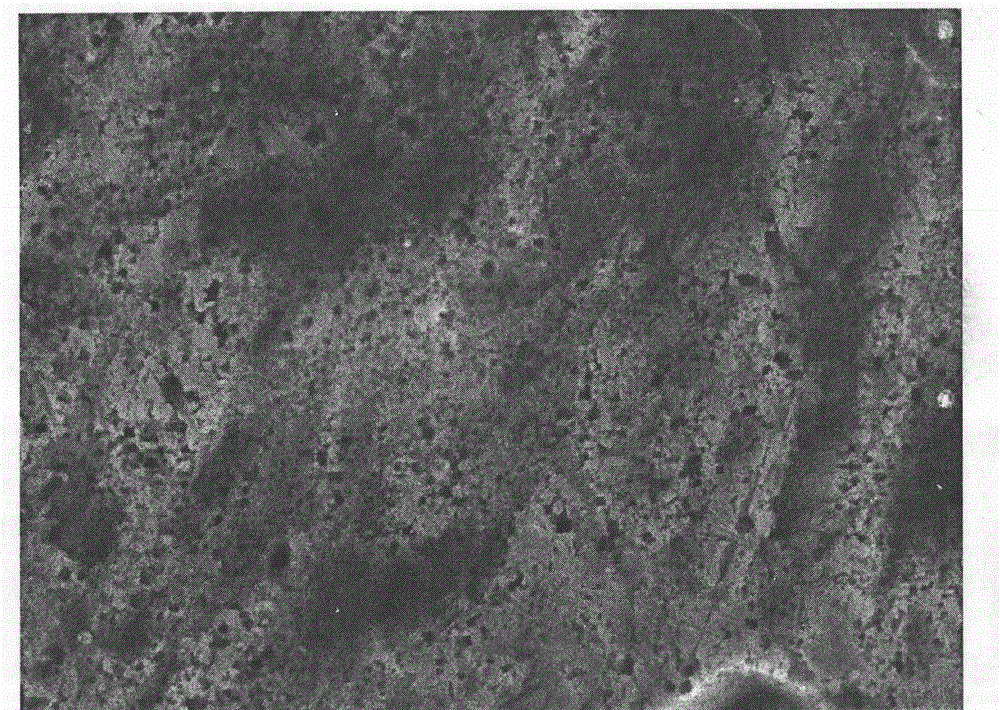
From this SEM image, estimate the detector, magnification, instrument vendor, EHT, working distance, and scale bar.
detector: SEI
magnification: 10 K X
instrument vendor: JEOL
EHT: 15 kV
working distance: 12.6 mm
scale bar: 1000 nm